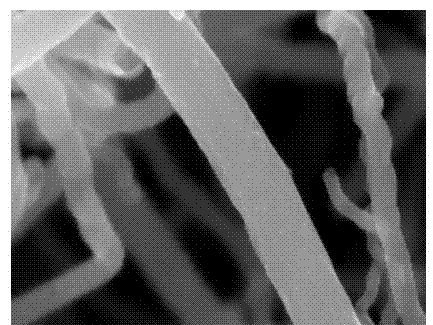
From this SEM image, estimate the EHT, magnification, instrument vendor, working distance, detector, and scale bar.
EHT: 5 kV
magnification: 150 K X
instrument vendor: Hitachi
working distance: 8.4 mm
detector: SE(M)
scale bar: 300 nm